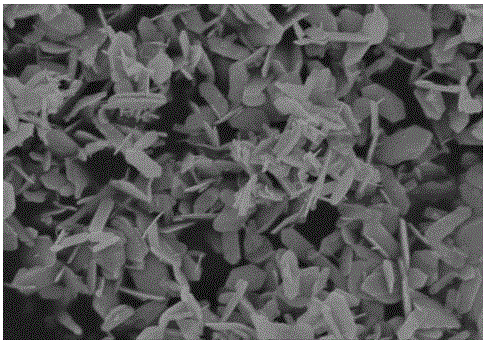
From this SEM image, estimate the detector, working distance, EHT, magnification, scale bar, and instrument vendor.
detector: SE(U,LA0)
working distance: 6.2 mm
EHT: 3 kV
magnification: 35 K X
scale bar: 1000 nm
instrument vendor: Hitachi